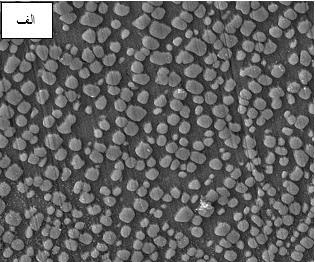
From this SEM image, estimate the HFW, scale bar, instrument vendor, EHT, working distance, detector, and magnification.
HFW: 13.8 µm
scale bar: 2000 nm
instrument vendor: Tescan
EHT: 15 kV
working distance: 9.99 mm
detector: SE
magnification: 15 K X